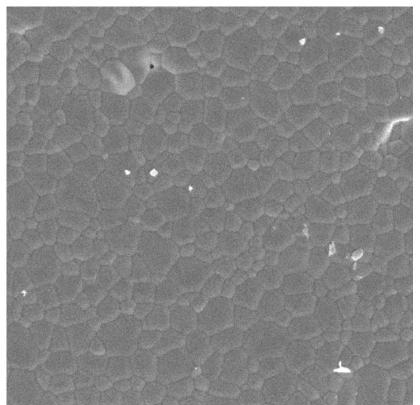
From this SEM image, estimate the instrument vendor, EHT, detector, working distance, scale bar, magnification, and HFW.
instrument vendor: Tescan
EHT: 20 kV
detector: SE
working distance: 11.16 mm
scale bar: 20000 nm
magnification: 2 K X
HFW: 142 µm